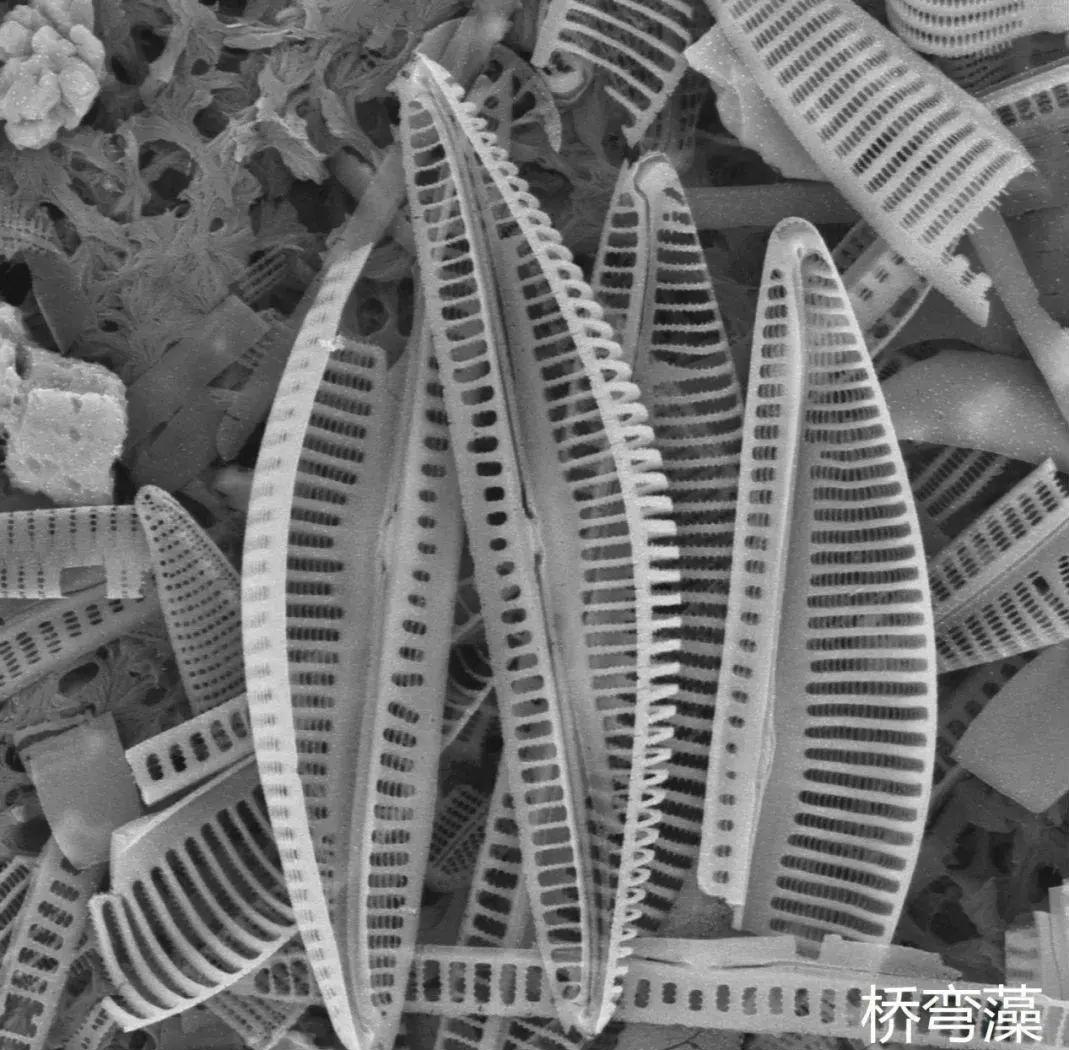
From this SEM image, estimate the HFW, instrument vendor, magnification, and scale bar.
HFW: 26.9 µm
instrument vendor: other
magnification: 10 K X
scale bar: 8000 nm